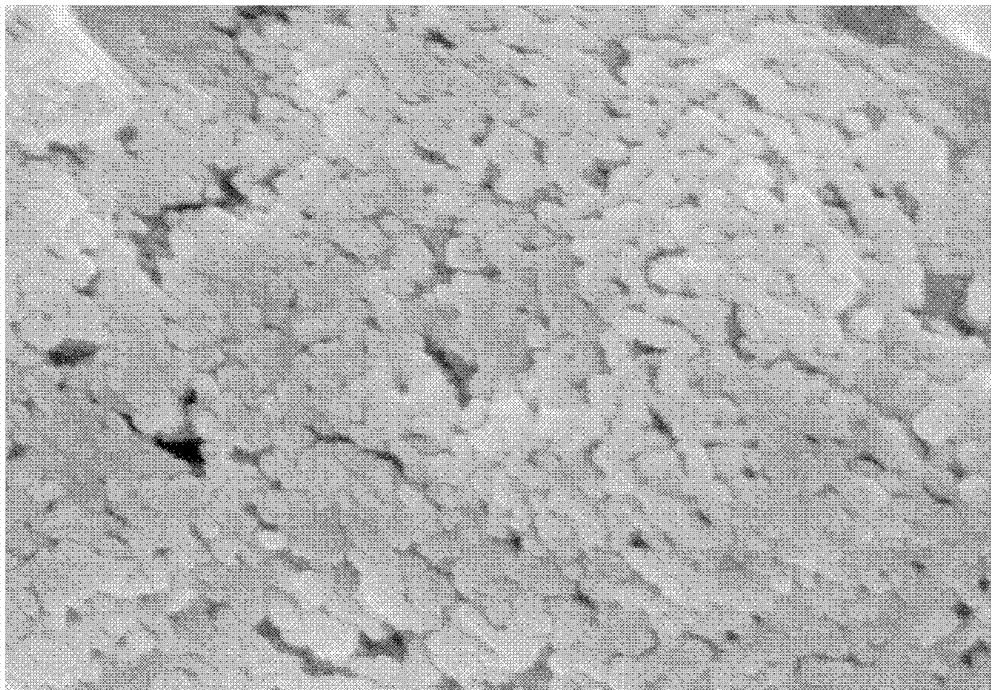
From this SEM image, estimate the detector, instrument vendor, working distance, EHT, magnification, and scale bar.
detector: SE(M)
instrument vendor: Hitachi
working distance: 7.8 mm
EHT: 5 kV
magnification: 80 K X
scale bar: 500 nm